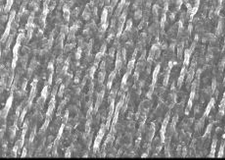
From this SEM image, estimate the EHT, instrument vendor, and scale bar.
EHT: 25 kV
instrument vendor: JEOL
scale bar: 100000 nm